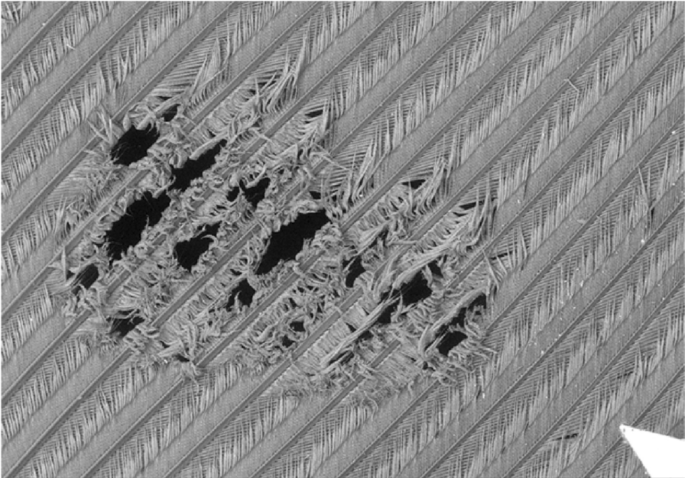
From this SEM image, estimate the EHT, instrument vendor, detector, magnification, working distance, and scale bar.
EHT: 15 kV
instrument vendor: Hitachi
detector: BSE3D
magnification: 0.025 K X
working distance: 32.9 mm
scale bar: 2000000 nm